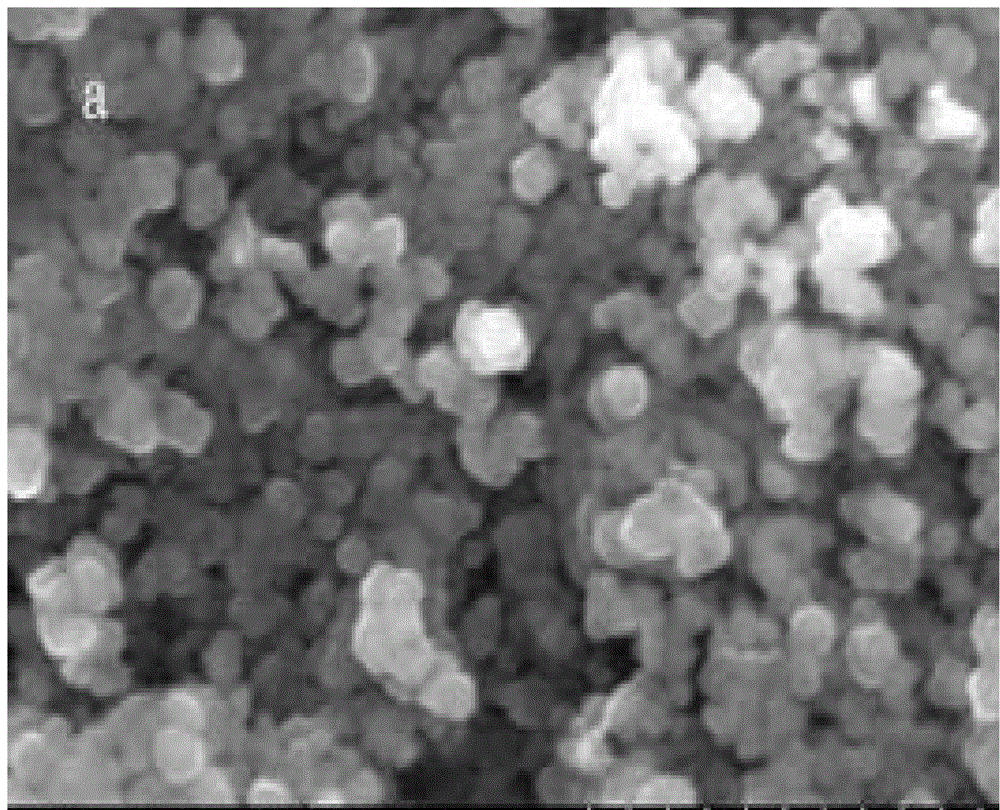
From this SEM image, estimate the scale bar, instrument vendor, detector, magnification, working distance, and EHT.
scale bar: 1000 nm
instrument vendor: Hitachi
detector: SE(M)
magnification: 50 K X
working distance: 19.4 mm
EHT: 15 kV